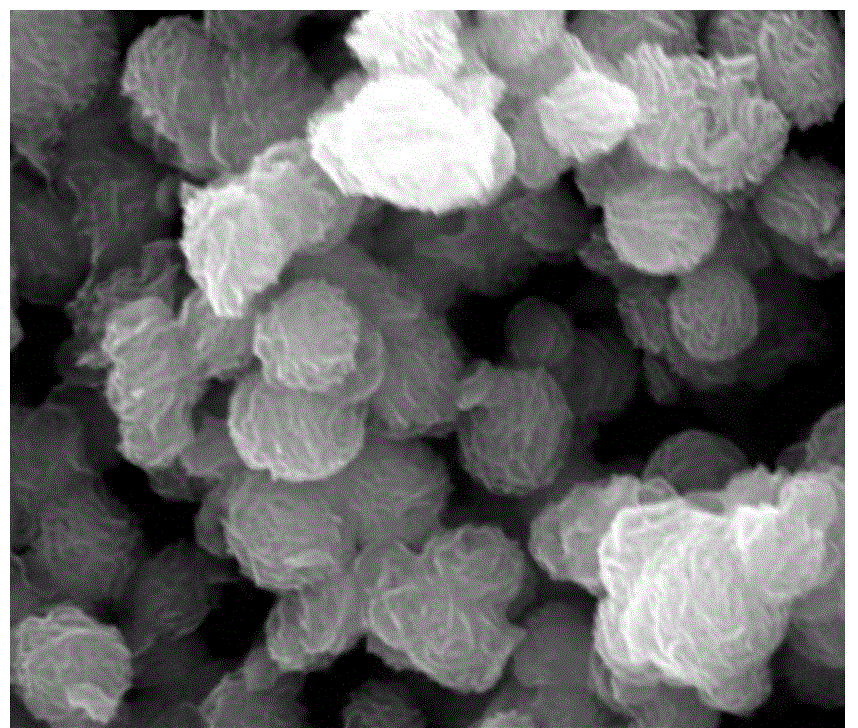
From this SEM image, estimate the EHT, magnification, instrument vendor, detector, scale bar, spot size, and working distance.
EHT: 20 kV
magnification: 100 K X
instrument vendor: FEI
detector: ETD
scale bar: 500 nm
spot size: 3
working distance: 10 mm